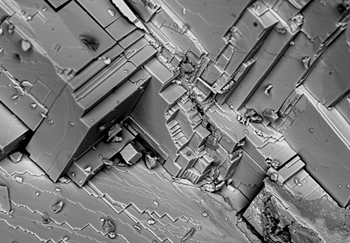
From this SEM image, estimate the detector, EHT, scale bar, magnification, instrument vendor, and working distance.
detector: BSECOMP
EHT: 25 kV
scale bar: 100000 nm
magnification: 0.5 K X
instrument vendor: Hitachi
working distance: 12.9 mm